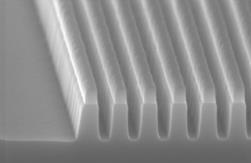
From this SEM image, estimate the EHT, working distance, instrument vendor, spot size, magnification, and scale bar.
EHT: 5 kV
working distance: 3.2 mm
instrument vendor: FEI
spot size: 3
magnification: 50 K X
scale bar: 500 nm